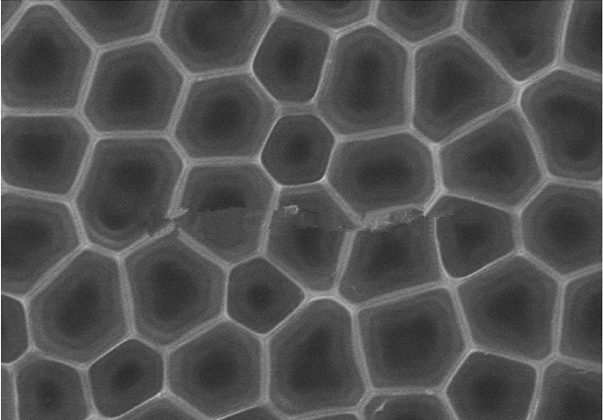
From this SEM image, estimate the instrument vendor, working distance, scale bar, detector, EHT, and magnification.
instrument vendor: Hitachi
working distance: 9.2 mm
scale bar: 1000 nm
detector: SE(M,LA0)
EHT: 20 kV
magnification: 50 K X